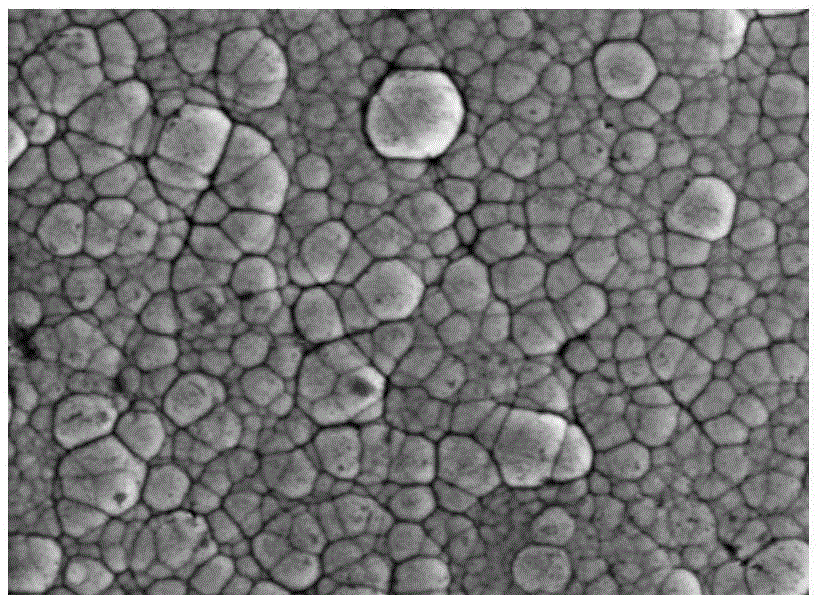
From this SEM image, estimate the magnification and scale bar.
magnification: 2 K X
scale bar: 5000 nm